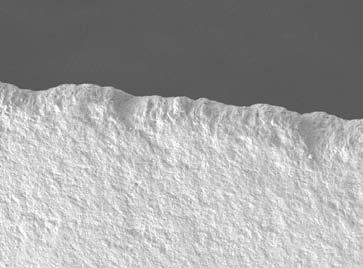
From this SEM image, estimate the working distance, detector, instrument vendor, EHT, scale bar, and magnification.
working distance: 9.1 mm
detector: LEI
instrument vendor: JEOL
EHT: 5 kV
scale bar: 10000 nm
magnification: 0.3 K X